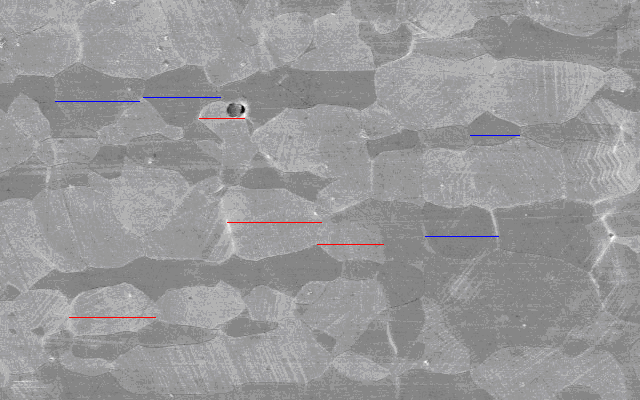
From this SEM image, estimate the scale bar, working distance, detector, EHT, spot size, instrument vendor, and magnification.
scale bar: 10000 nm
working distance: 16 mm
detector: SE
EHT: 15 kV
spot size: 4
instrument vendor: FEI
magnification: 1.5 K X